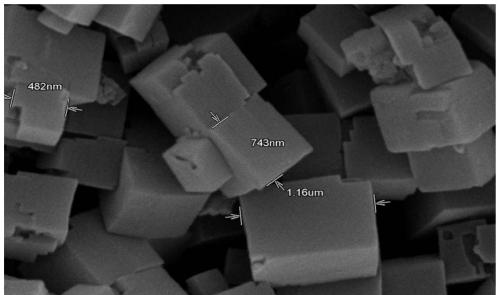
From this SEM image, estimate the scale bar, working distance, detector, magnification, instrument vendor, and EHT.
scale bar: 1000 nm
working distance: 5 mm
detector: SE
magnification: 30 K X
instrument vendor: Hitachi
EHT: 15 kV